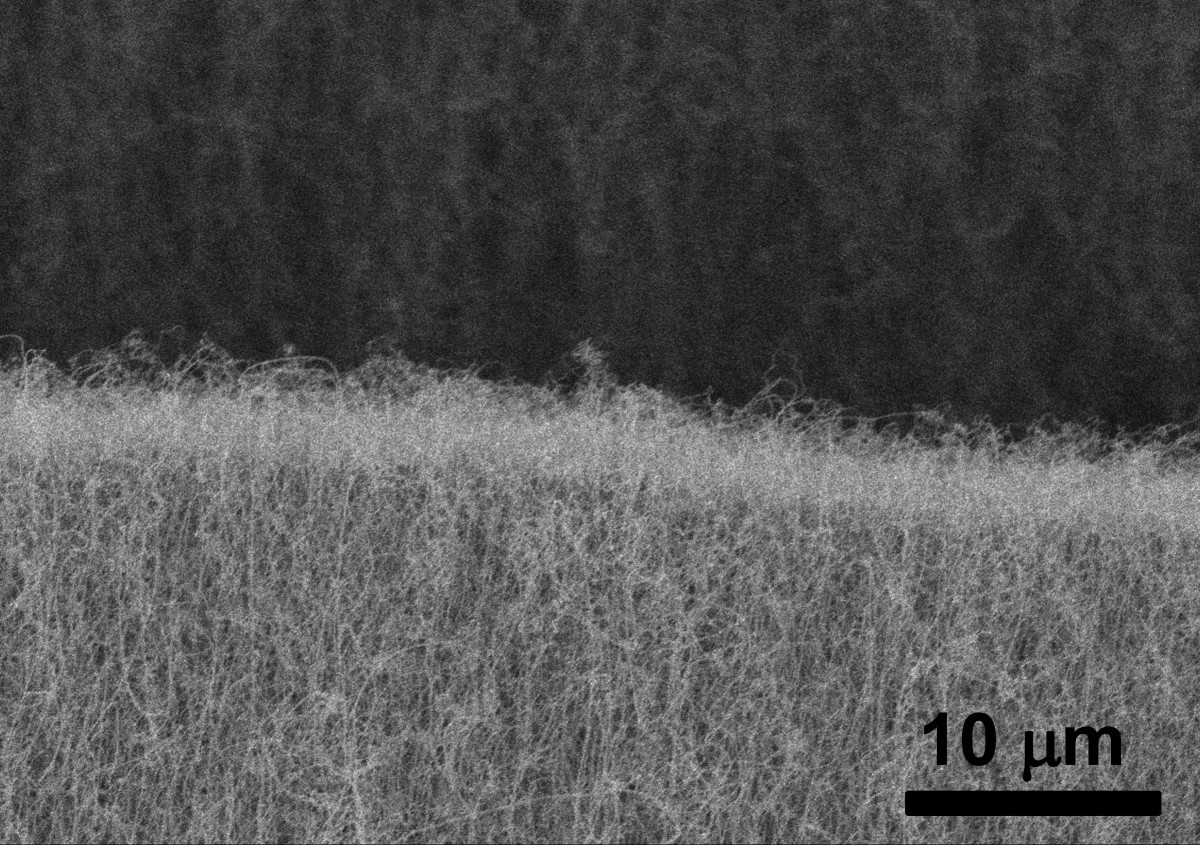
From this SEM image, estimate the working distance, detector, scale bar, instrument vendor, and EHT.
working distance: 14.6 mm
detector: SEI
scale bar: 10000 nm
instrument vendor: JEOL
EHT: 10 kV